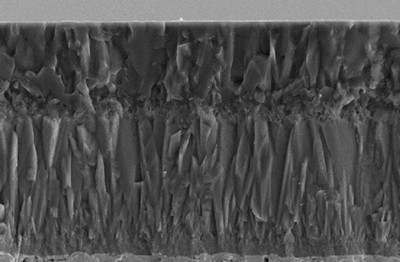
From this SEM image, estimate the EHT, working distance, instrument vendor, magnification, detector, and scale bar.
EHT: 10 kV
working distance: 12.2 mm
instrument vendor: Zeiss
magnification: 4.5 K X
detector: SE2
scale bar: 1000 nm